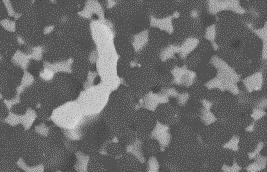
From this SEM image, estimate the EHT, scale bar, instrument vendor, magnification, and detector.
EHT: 20 kV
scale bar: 5000 nm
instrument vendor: JEOL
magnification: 5.3 K X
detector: BES